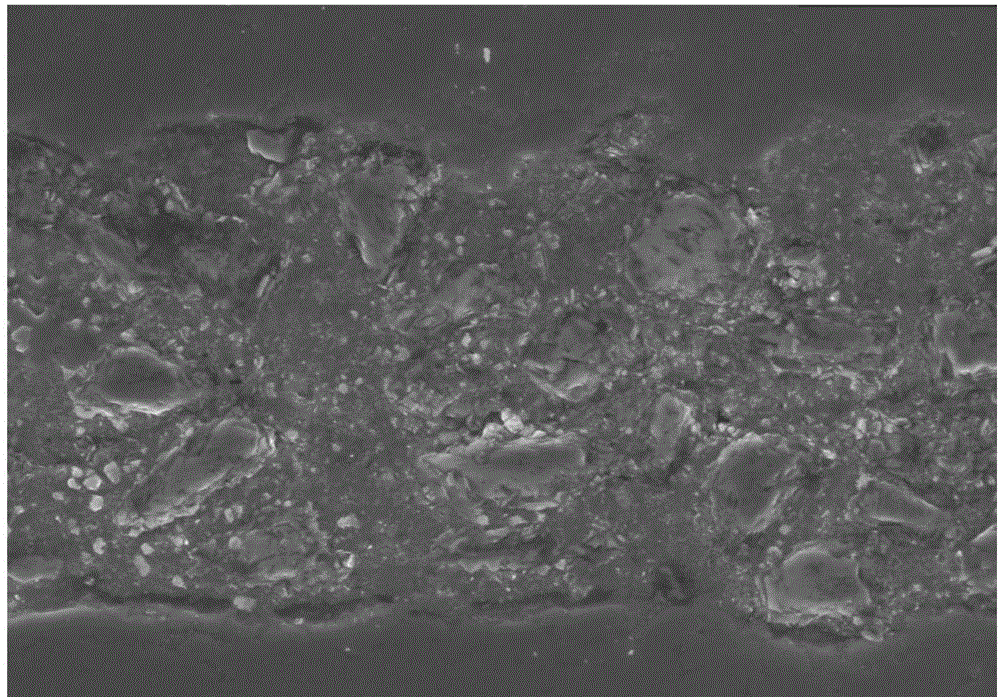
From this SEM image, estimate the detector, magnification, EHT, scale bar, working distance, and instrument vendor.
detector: SE(U)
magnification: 1.2 K X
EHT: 15 kV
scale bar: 40000 nm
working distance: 16.4 mm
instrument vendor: Hitachi